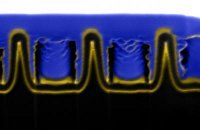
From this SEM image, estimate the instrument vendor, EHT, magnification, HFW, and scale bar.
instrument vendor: other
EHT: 30 kV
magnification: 30 K X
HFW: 4.27 µm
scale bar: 500 nm